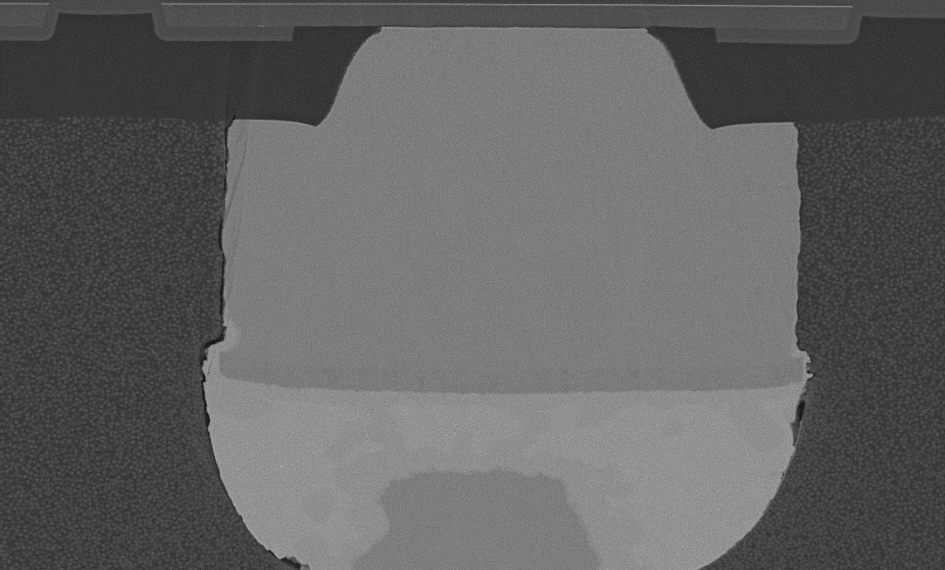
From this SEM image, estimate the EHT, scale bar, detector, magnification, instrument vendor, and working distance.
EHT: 10 kV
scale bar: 40000 nm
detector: LA100(U)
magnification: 1.3 K X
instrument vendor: Hitachi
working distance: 8 mm